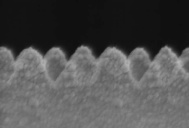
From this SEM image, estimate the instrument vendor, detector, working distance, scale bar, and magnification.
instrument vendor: Hitachi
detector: SE(M)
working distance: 8.6 mm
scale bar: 500 nm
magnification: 90 K X